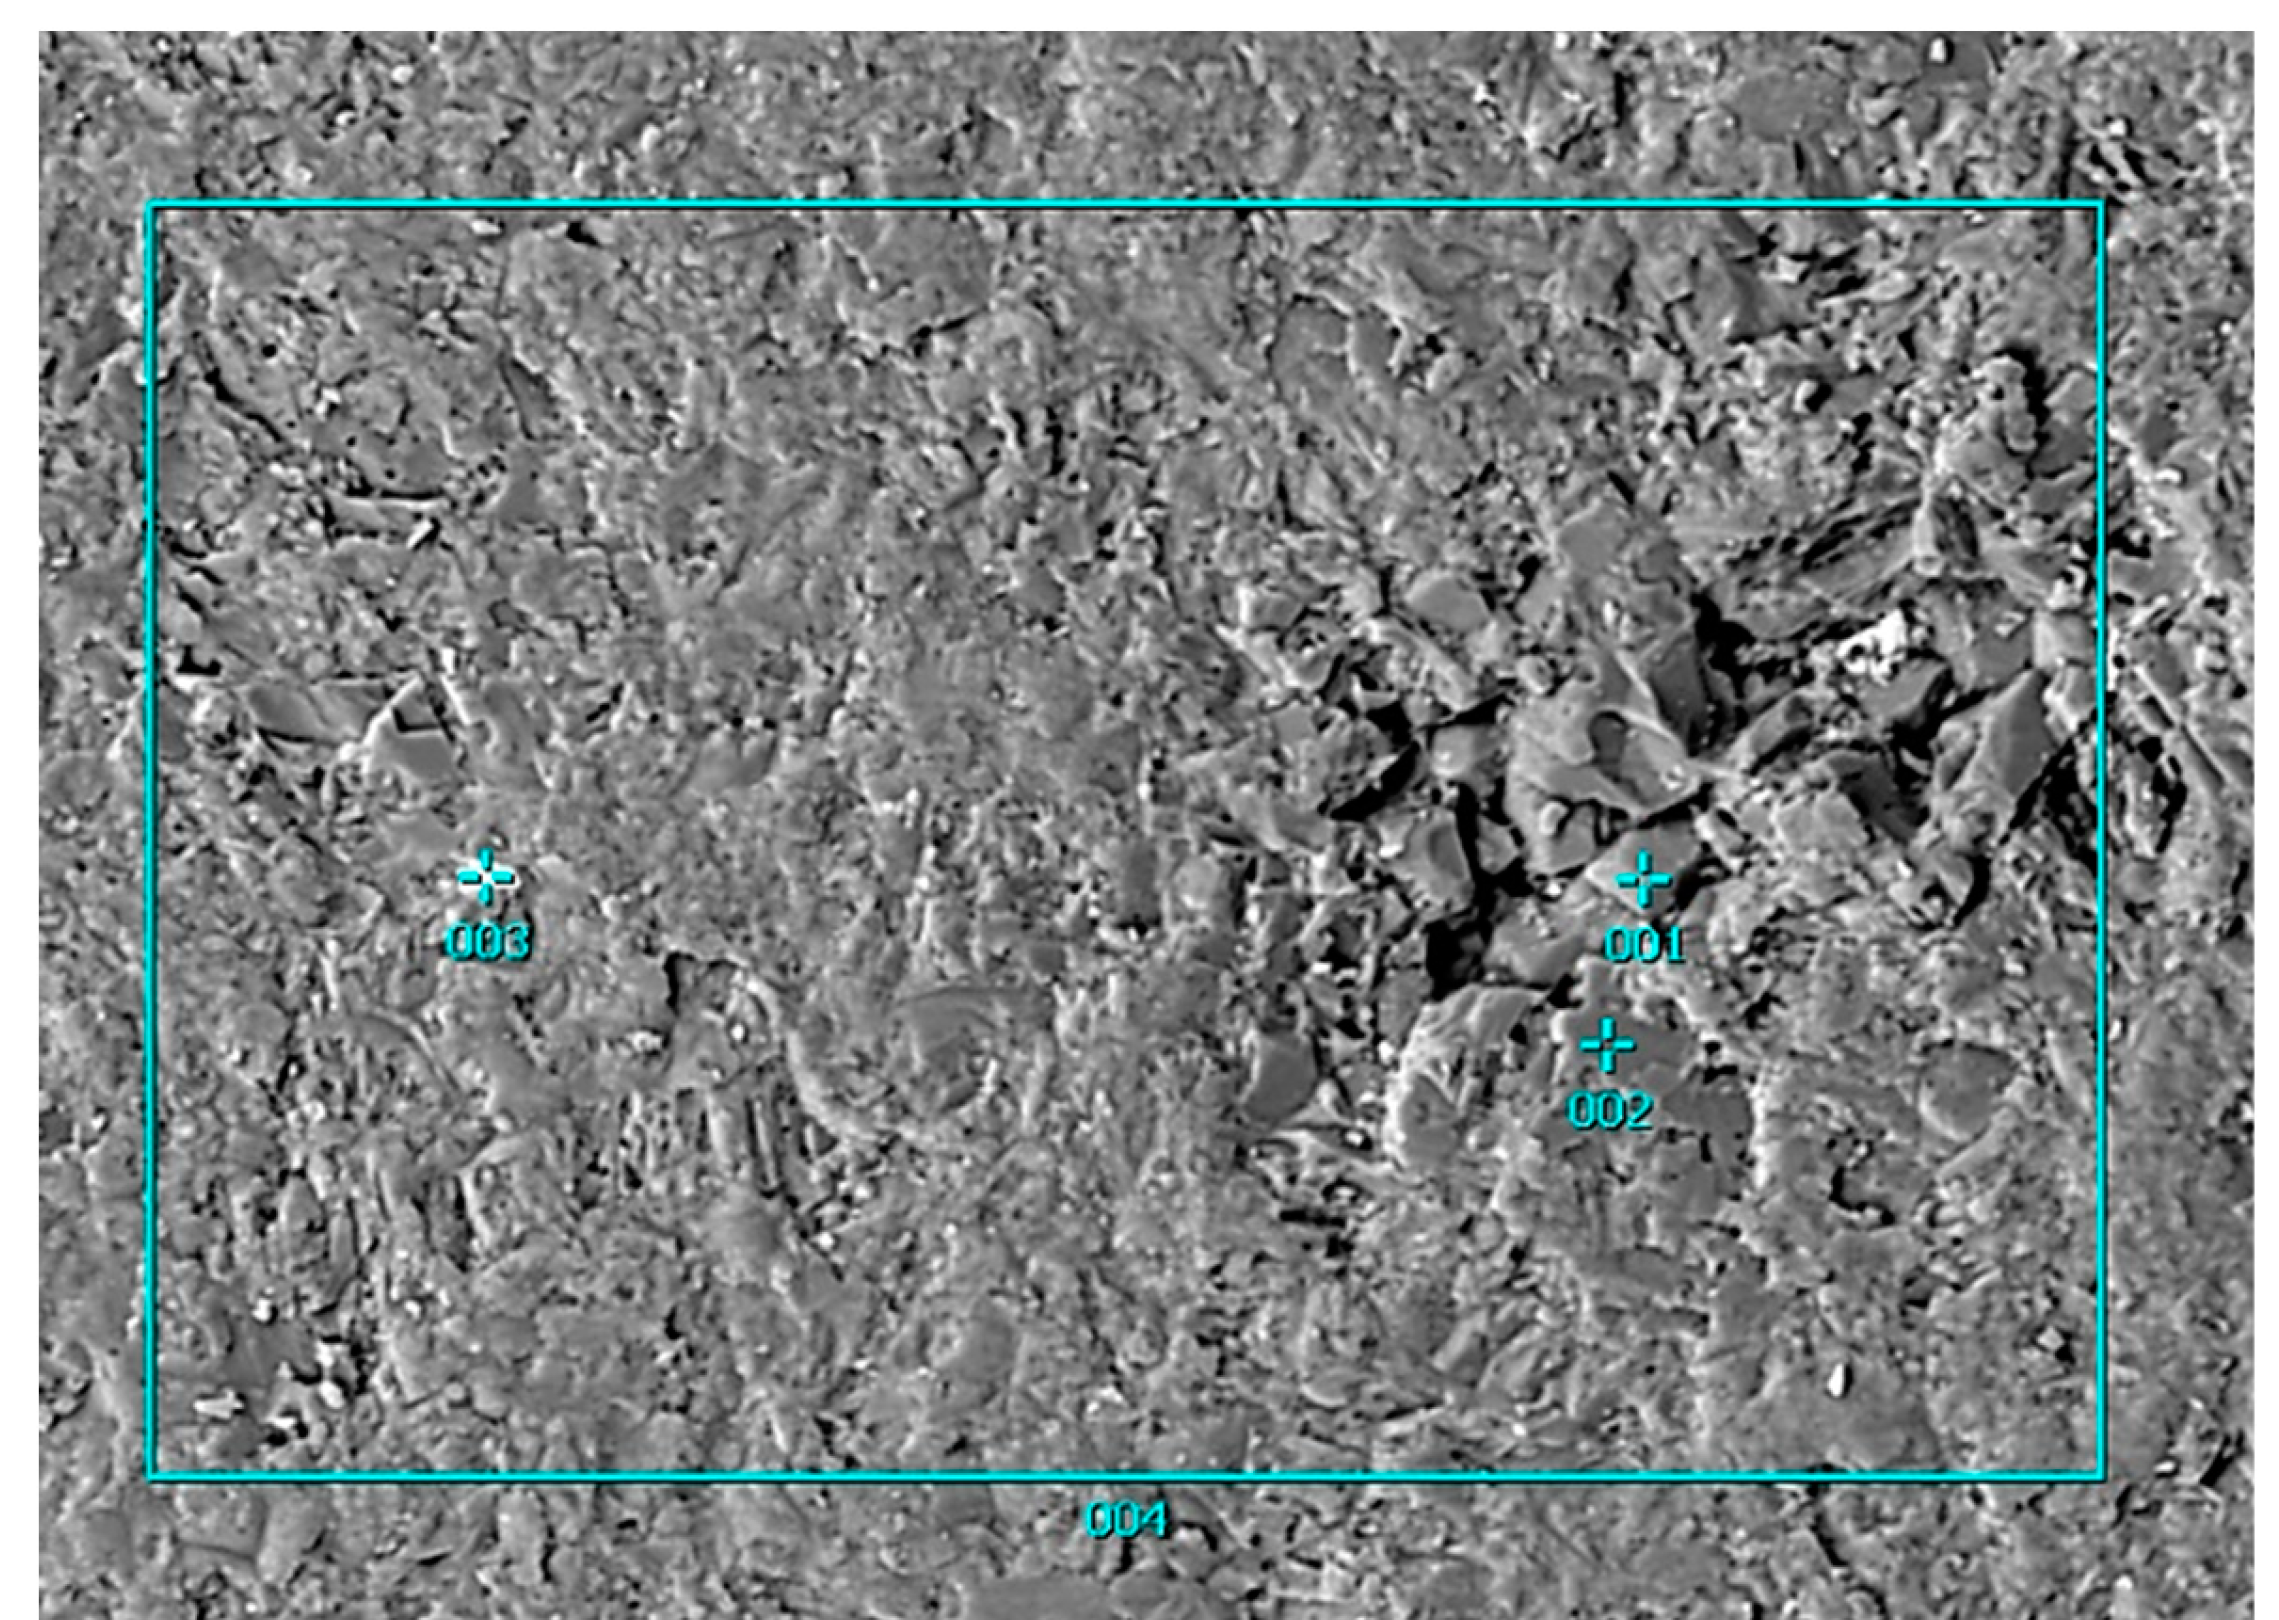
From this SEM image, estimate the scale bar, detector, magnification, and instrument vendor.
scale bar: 10000 nm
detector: COMPO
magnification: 0.5 K X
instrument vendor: JEOL